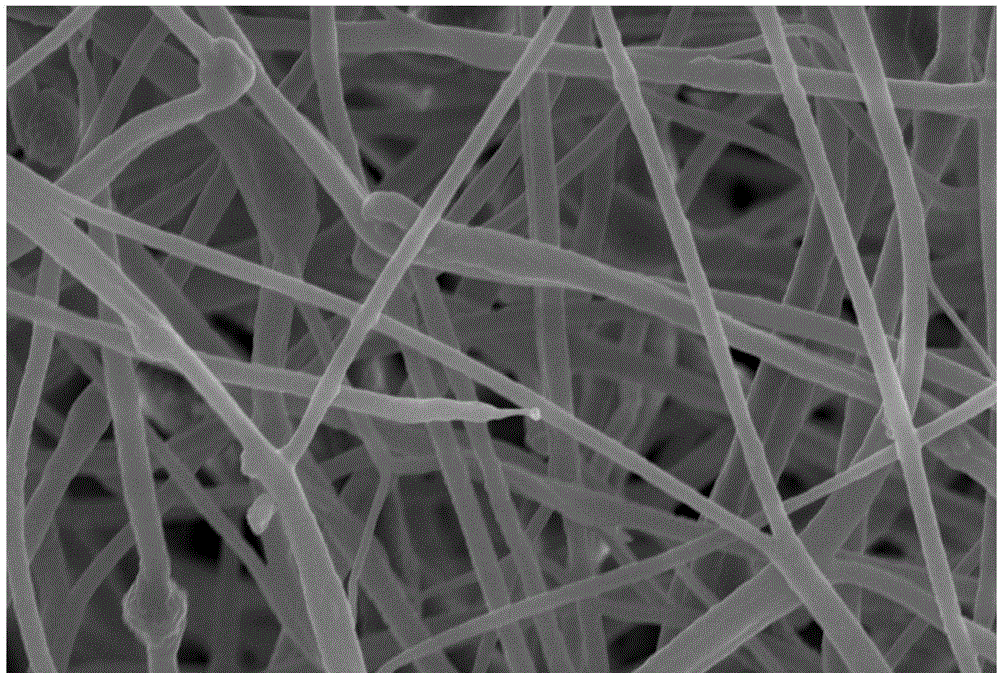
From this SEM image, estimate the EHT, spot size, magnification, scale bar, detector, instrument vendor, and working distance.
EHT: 15 kV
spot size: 50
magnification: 1.8 K X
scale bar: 10000 nm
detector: SEI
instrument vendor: JEOL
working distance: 8 mm